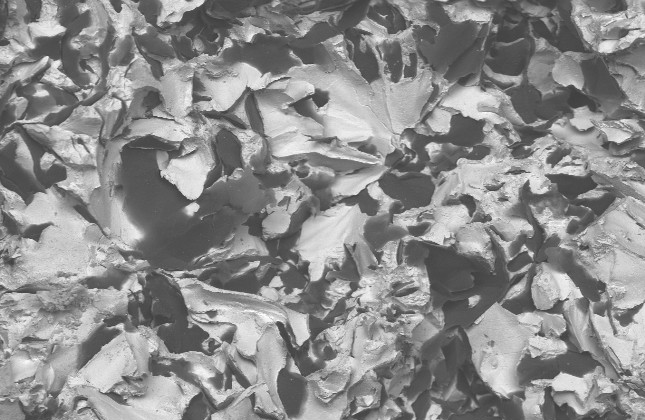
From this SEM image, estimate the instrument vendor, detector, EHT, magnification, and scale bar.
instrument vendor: FEI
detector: BSE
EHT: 20 kV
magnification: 0.1 K X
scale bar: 200000 nm